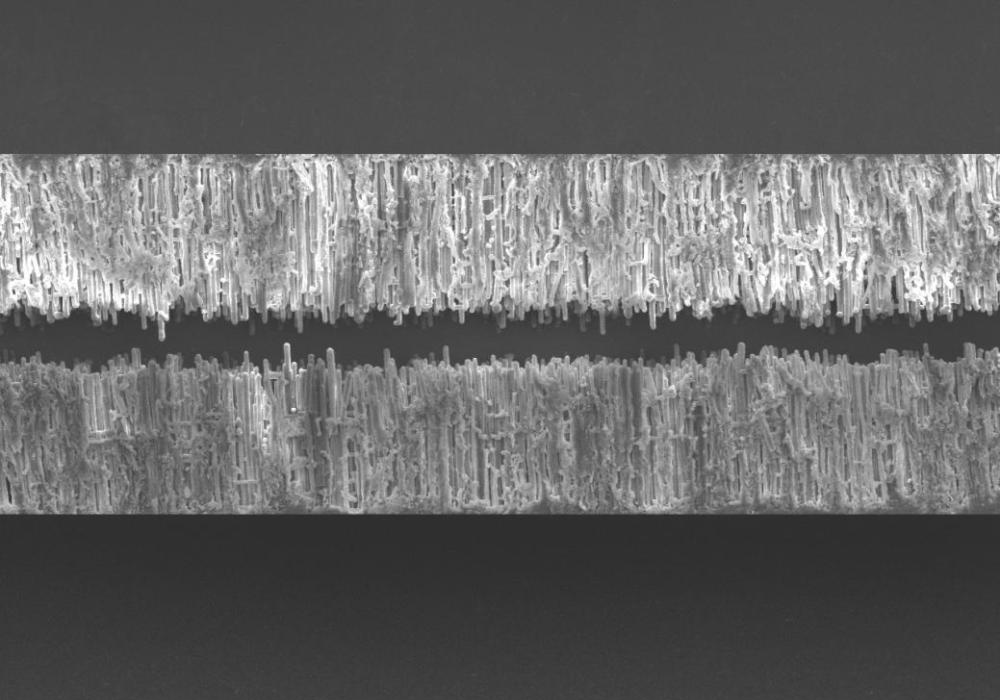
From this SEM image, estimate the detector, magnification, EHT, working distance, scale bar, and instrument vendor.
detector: SEI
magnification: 0.35 K X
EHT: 10 kV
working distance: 10 mm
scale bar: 10000 nm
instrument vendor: JEOL